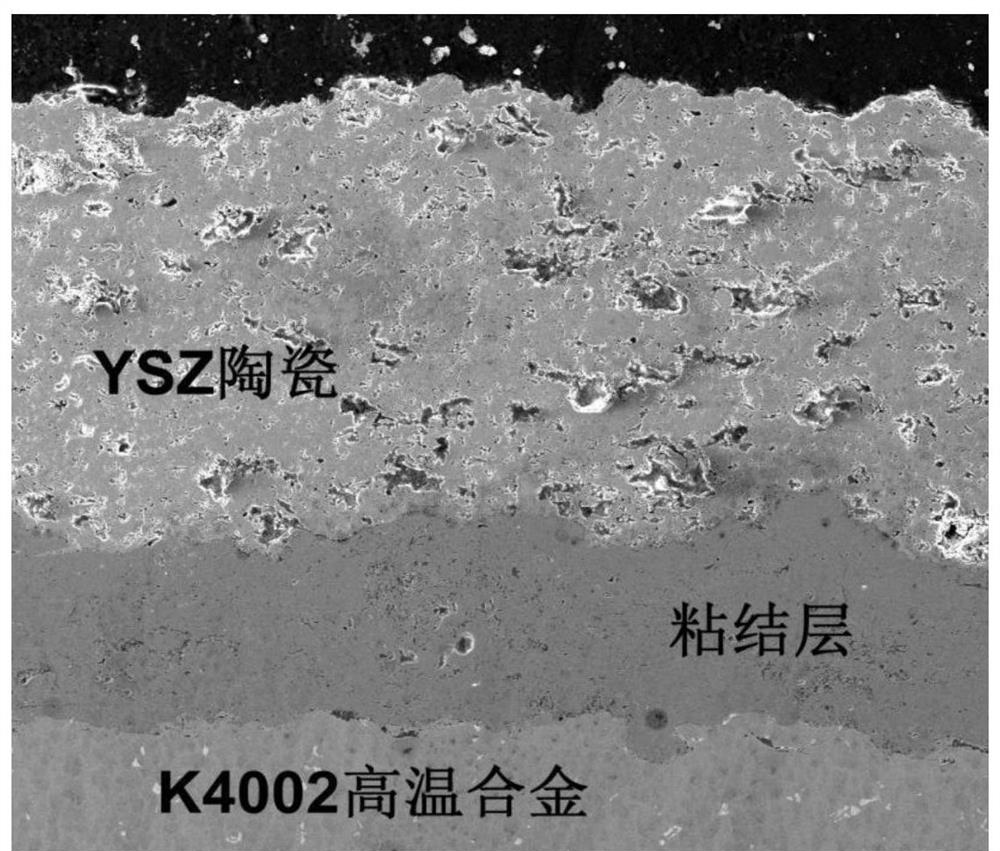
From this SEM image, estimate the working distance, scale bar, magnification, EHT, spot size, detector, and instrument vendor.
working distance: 11 mm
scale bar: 200000 nm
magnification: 0.5 K X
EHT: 14.98 kV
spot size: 4.5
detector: SE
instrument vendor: FEI